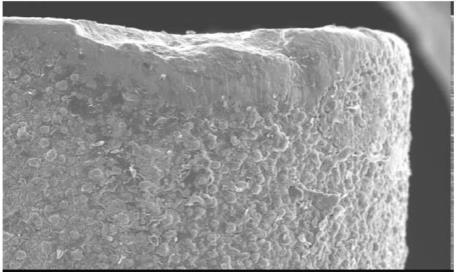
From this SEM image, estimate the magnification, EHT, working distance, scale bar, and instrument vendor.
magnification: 0.1 K X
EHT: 20 kV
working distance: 24 mm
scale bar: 100000 nm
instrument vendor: JEOL